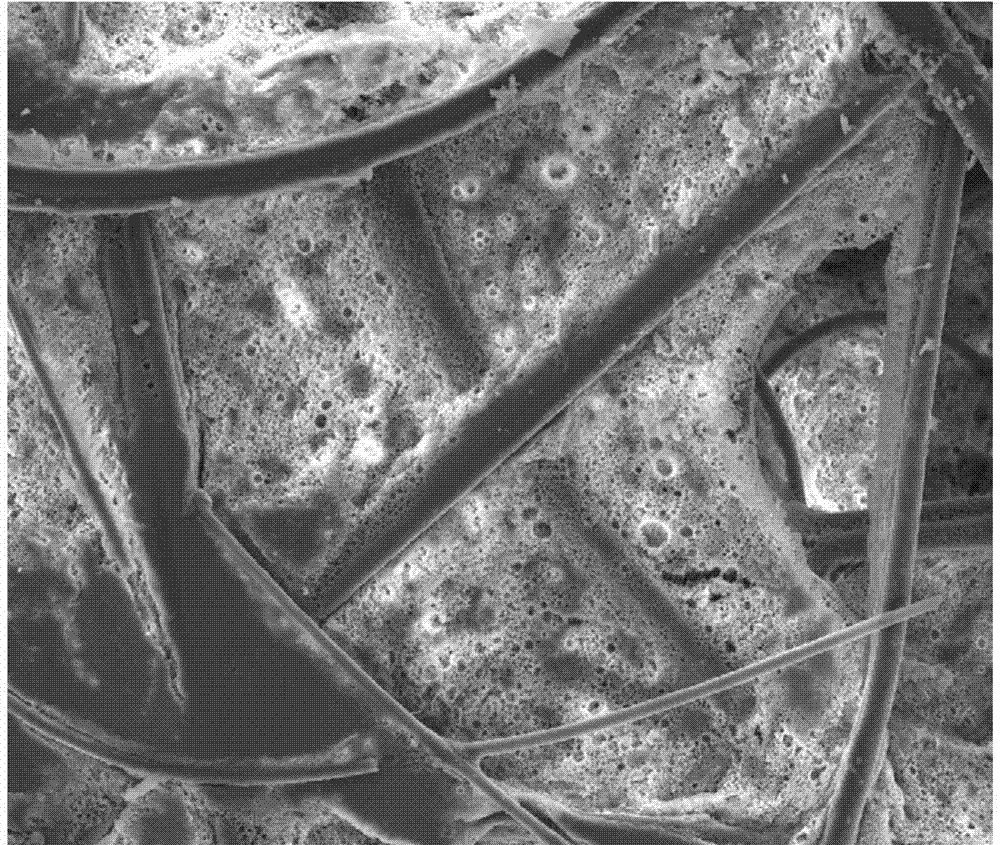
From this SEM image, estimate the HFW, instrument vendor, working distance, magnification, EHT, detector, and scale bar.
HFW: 423 µm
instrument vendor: FEI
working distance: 13.5 mm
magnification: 0.3 K X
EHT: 20 kV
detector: ETD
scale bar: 200000 nm